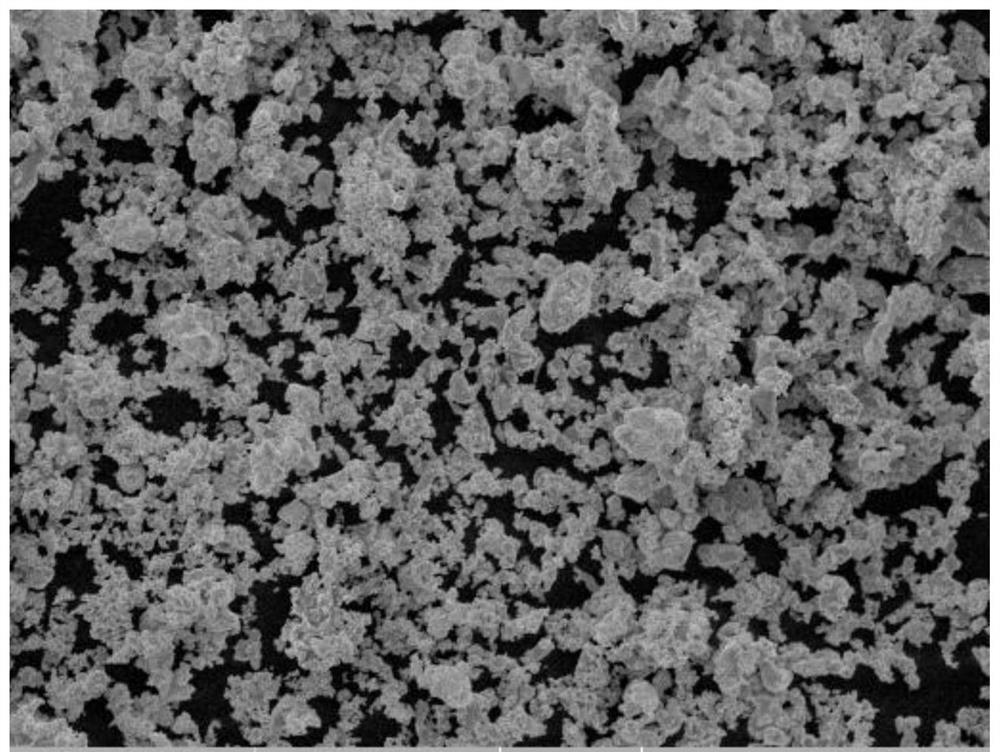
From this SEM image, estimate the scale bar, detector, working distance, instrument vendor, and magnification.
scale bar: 20000 nm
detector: SE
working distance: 11.7 mm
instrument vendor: Tescan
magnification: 2 K X